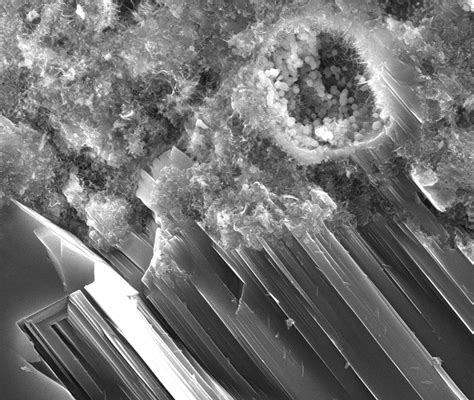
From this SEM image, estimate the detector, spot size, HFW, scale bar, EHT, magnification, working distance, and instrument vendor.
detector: ETD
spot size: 3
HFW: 34.1 µm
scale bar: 10000 nm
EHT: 20 kV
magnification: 7.5 K X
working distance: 14 mm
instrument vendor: FEI